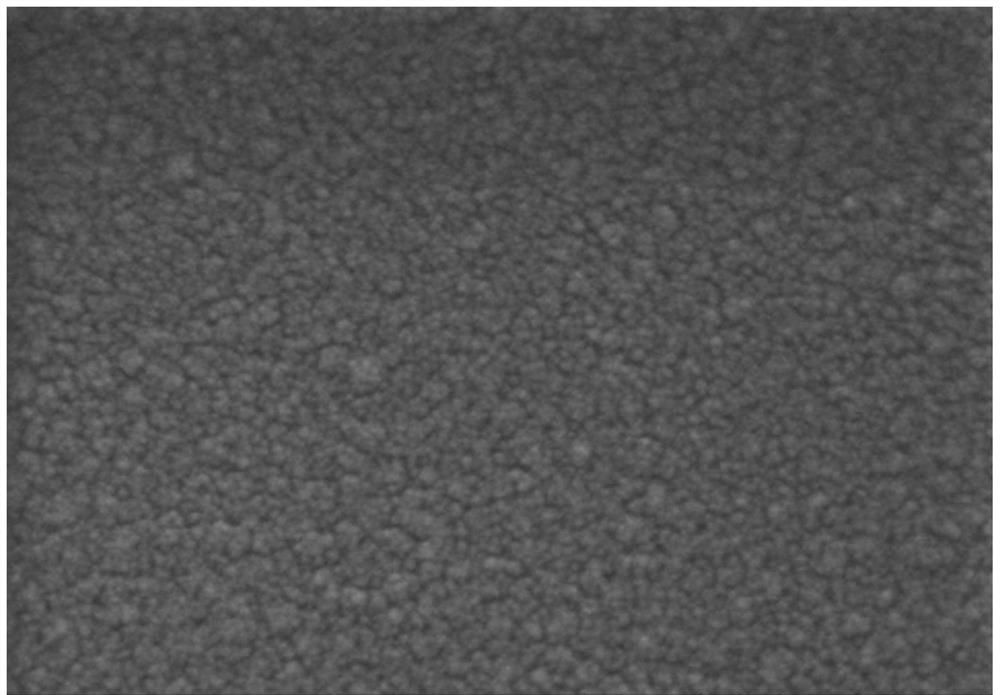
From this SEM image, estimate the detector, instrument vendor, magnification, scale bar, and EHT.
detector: SE(U)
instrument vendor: Hitachi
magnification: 100 K X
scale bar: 500 nm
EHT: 4 kV